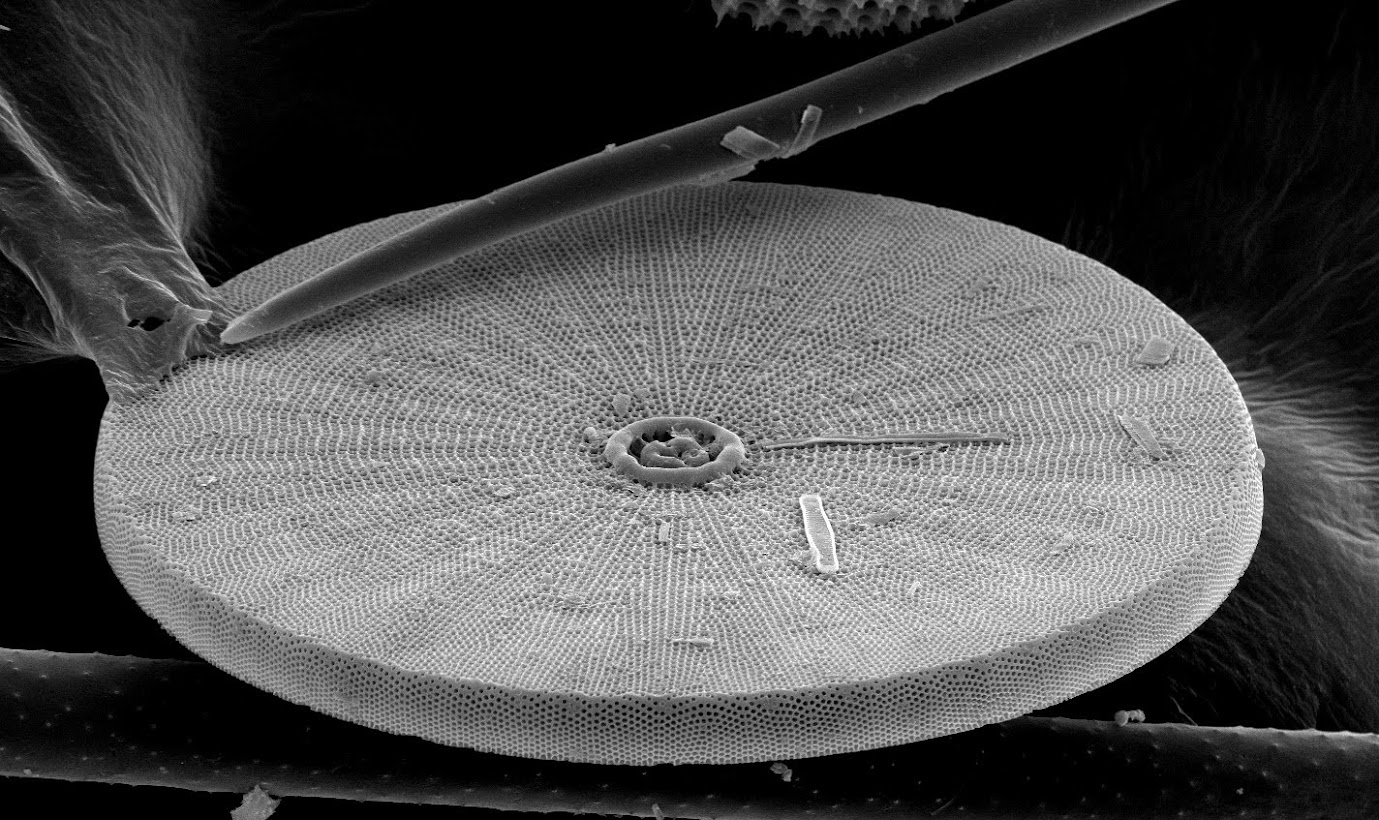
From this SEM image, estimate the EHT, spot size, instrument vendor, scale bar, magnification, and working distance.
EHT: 25 kV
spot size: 3.5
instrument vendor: FEI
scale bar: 50000 nm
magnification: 0.8 K X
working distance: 28.9 mm